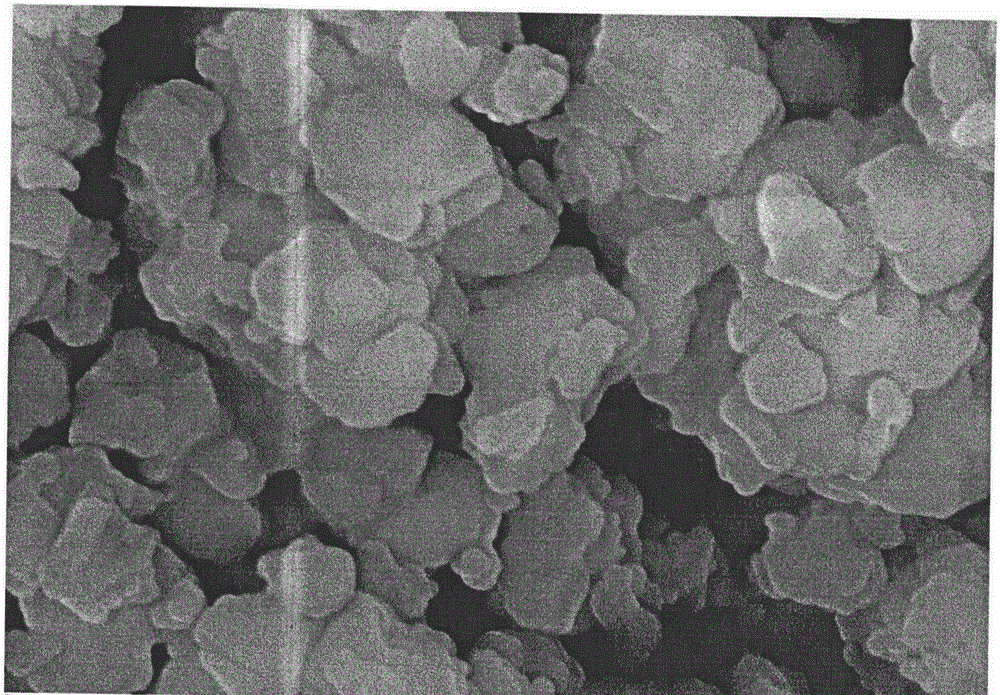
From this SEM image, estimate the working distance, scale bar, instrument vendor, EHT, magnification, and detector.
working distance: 6.6 mm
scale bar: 200 nm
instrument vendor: Zeiss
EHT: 10 kV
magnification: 50 K X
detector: InlensDuo